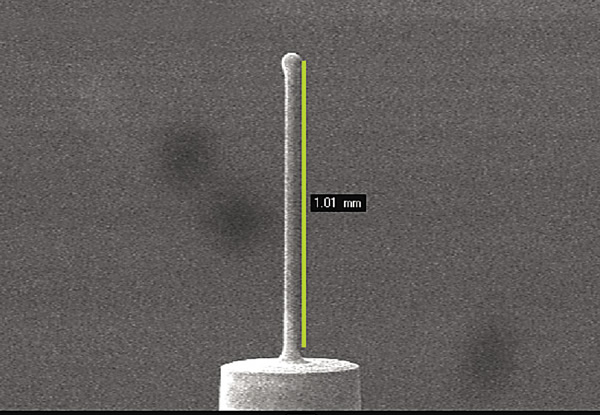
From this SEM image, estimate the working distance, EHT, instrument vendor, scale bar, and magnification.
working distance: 12 mm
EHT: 21 kV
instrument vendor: JEOL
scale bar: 100000 nm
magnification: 0.0488 K X